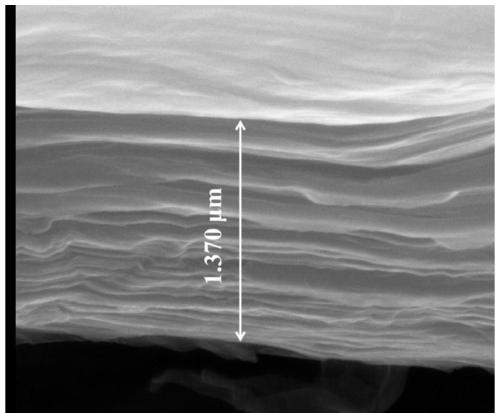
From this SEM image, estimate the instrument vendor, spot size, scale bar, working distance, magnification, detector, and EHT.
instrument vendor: FEI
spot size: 3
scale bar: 1000 nm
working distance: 11.1 mm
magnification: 100 K X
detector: ETD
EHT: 30 kV